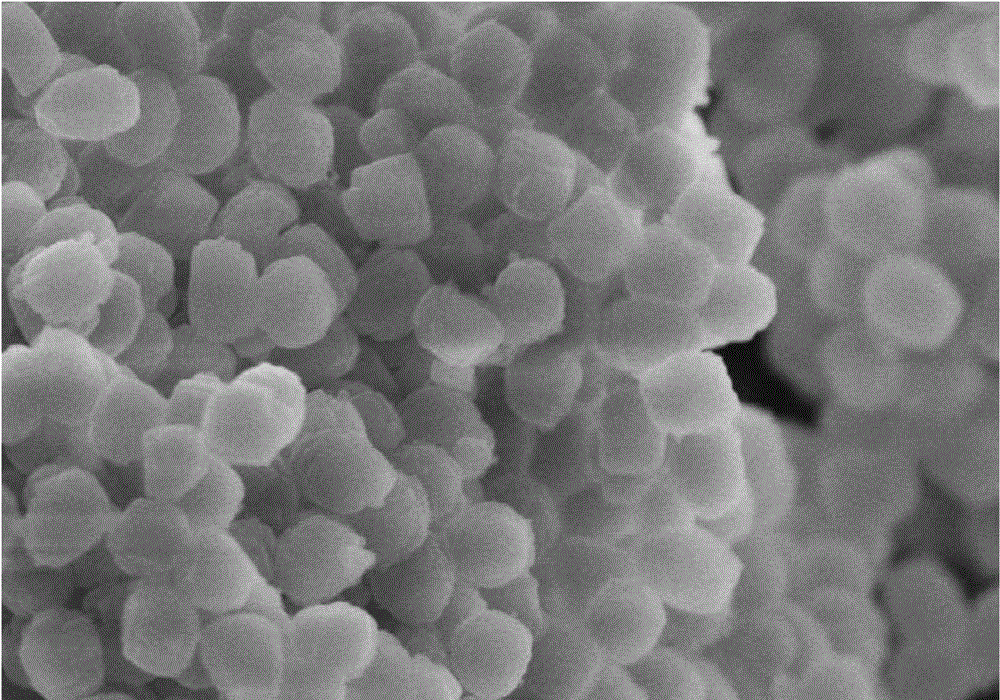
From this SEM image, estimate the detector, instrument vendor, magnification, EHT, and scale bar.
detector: SE(M)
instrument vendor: Hitachi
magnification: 30 K X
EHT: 5 kV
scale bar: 1000 nm